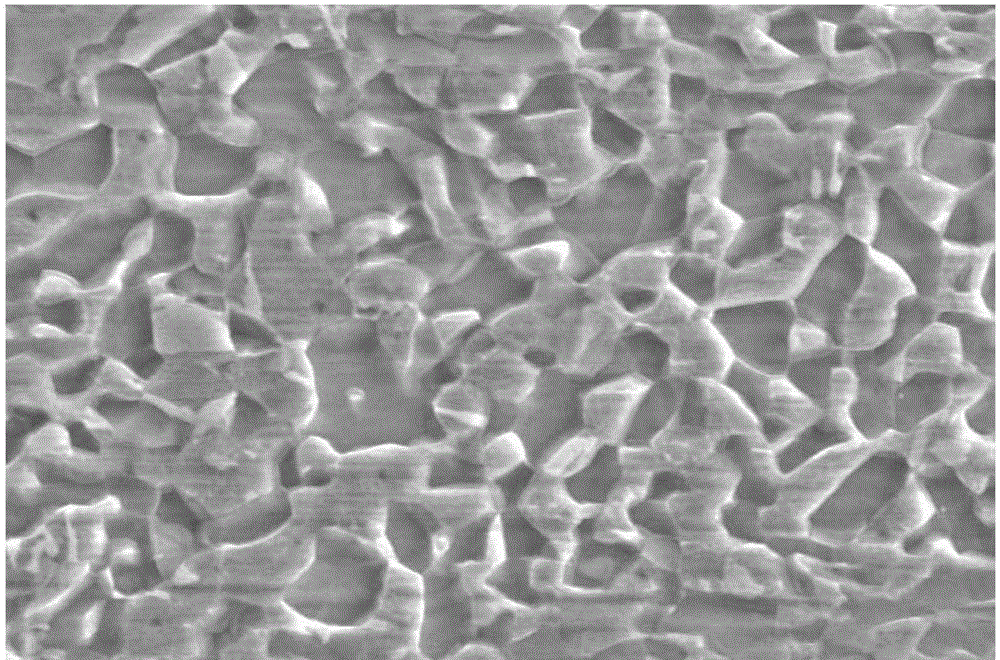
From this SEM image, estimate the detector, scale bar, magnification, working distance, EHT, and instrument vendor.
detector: SE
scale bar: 8000 nm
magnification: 5 K X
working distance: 11.6 mm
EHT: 15 kV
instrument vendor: Hitachi